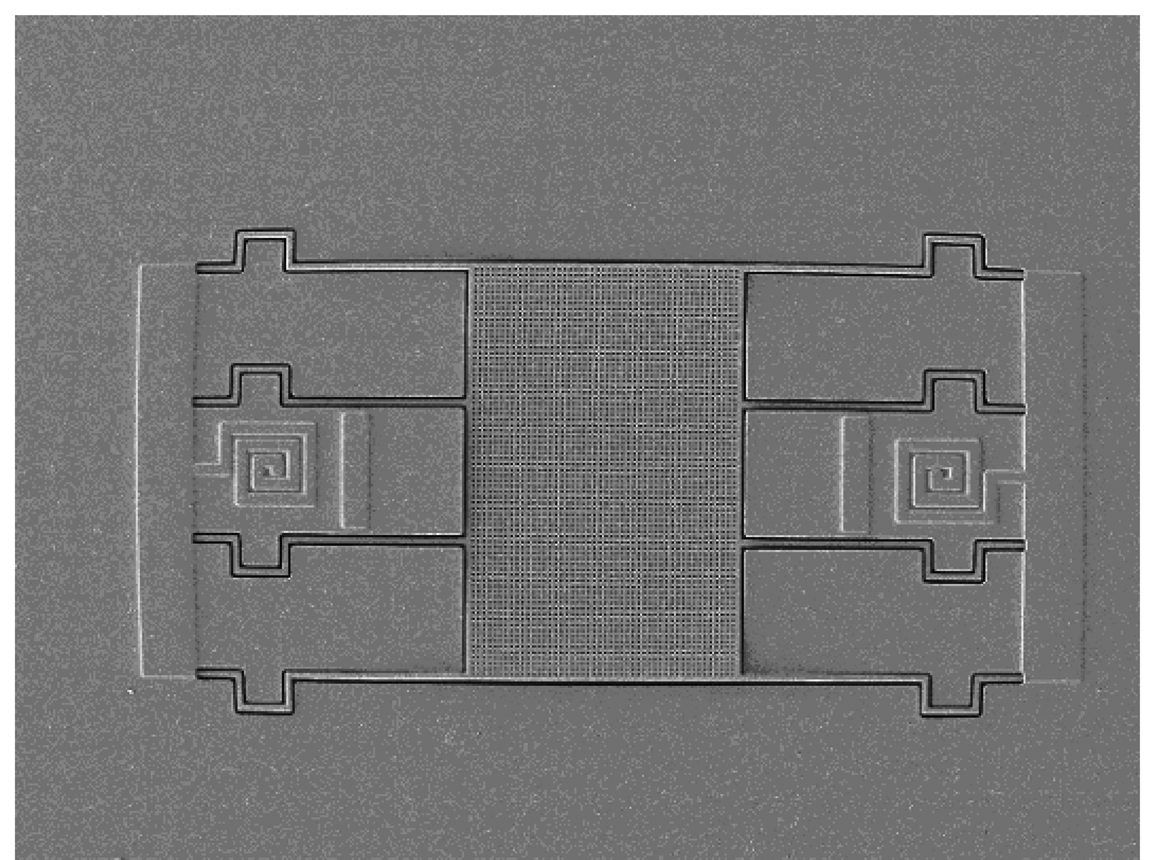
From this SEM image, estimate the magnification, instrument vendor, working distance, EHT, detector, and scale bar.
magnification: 0.3 K X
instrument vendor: JEOL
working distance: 7.6 mm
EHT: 3 kV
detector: LEI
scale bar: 10000 nm